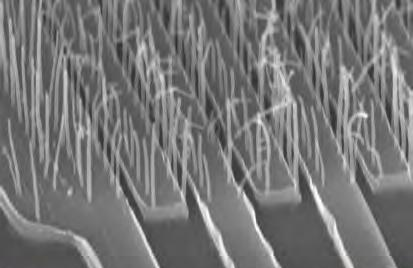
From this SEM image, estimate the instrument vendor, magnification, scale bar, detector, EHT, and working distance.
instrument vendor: Zeiss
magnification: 61.91 K X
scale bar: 300 nm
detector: InLens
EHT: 15 kV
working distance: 3.9 mm